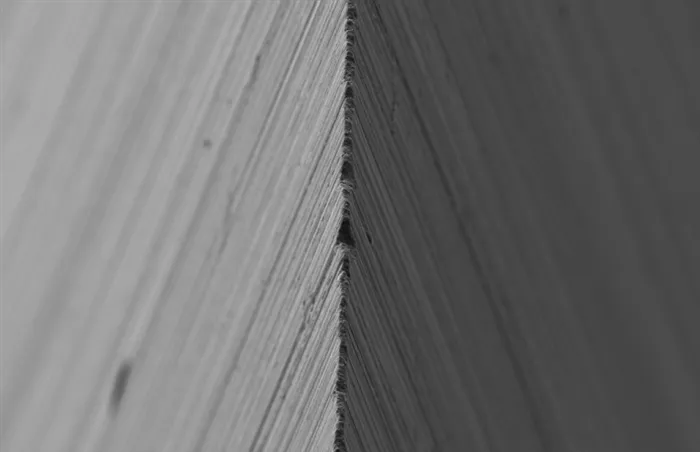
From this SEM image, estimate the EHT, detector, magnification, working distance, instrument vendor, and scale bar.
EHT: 5 kV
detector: SE2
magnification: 1 K X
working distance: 6.5 mm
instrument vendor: Zeiss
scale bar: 10000 nm